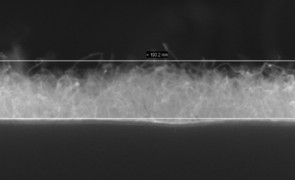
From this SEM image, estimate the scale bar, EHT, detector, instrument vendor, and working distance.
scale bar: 100 nm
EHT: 10 kV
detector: InLens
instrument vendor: Zeiss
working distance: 3 mm